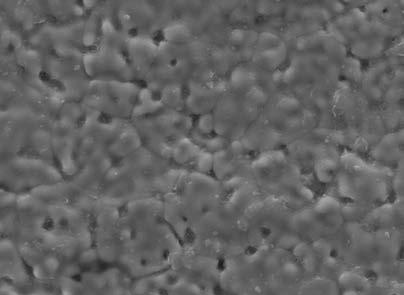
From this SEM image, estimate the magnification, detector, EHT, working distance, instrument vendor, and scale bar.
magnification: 3 K X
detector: LED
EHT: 20 kV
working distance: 12.5 mm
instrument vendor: JEOL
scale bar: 1000 nm